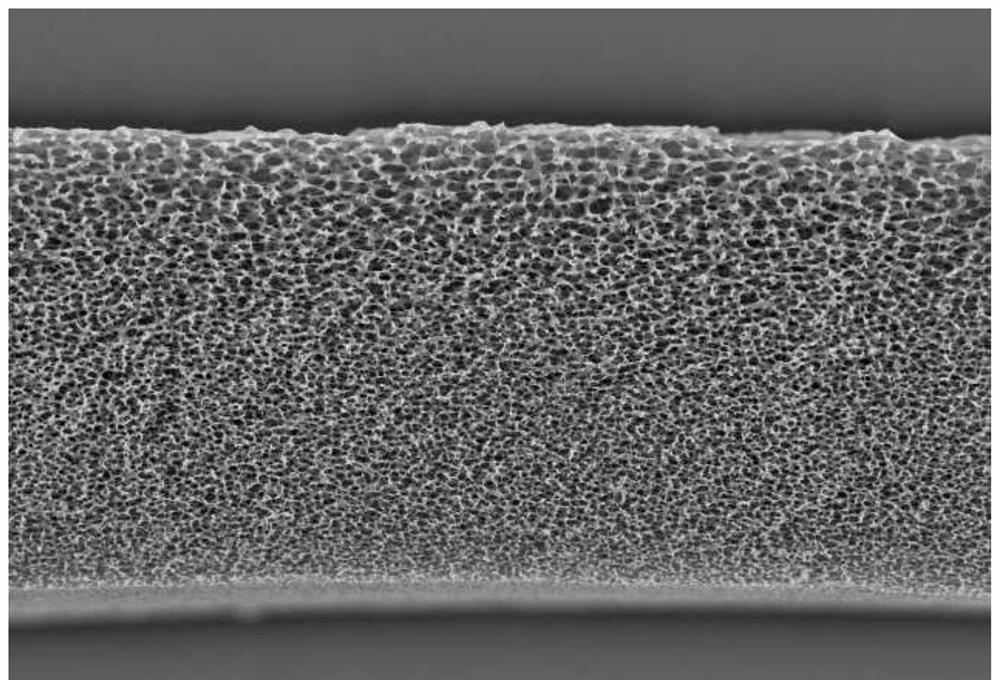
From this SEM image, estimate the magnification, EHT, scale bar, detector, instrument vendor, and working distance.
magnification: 0.5 K X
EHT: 20 kV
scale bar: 20000 nm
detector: S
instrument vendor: Hitachi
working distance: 7.8 mm